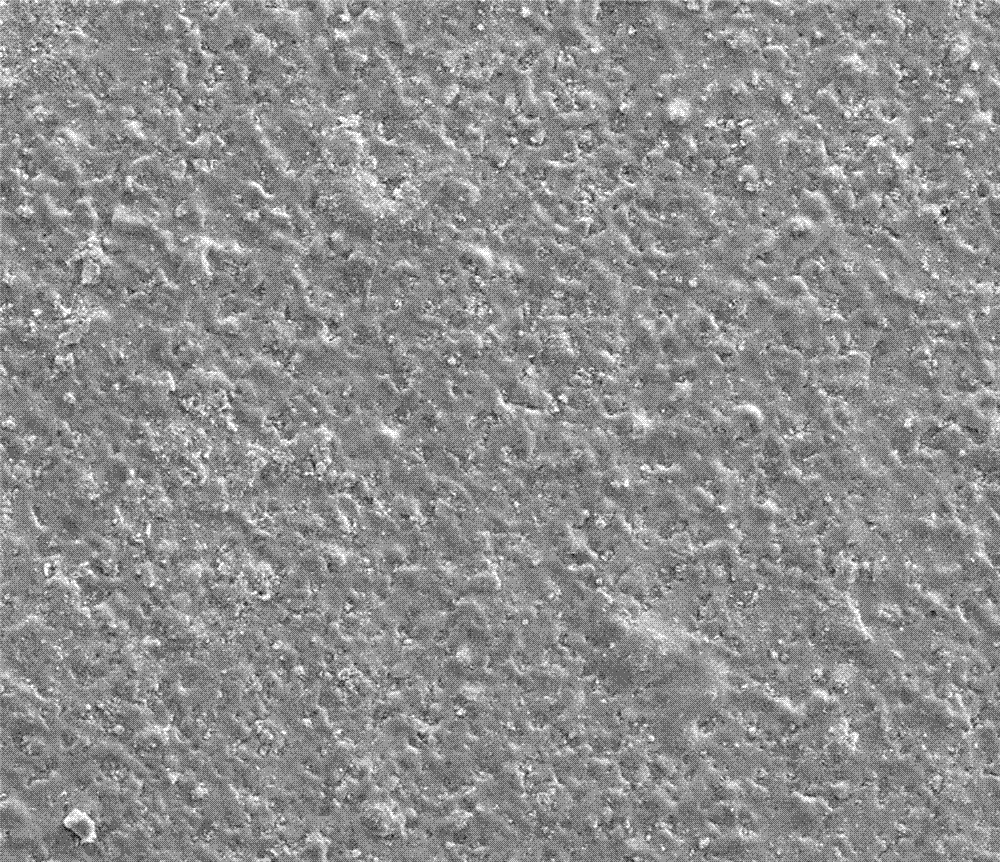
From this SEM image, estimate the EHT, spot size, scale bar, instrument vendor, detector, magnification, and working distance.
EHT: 20 kV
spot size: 4.5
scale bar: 300000 nm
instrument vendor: FEI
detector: ETD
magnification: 0.5 K X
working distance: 9.5 mm